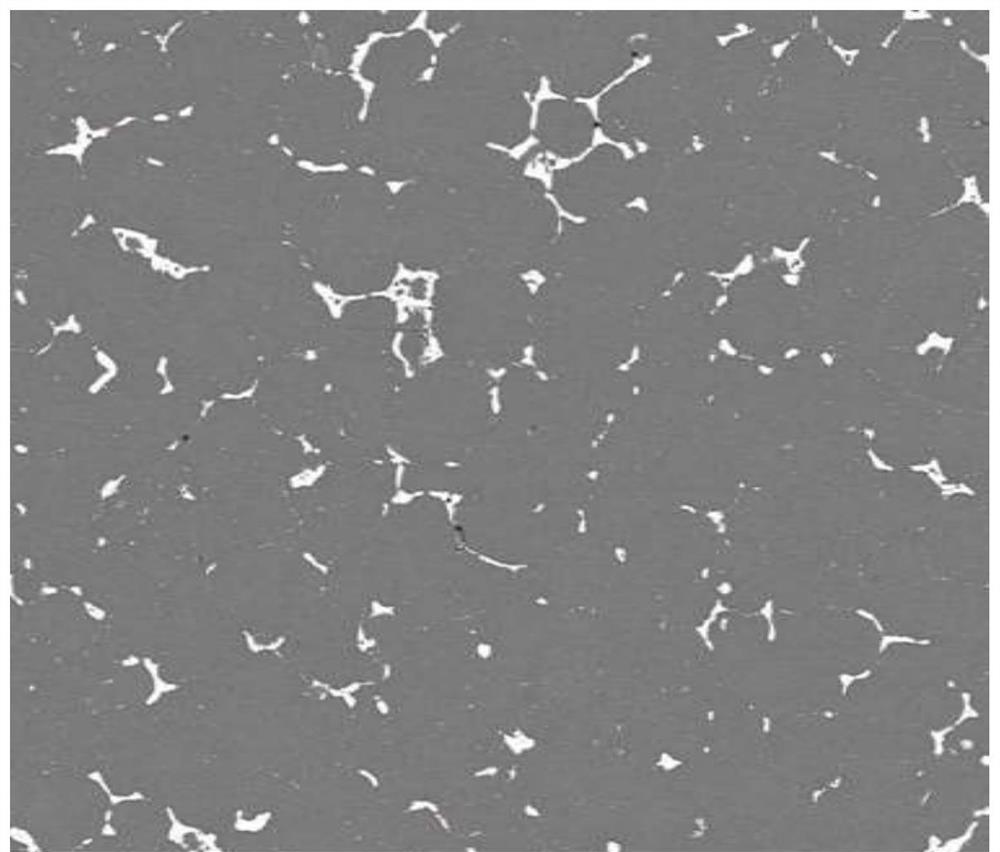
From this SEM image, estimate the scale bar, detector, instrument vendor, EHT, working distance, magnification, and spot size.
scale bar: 200000 nm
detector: DualBSD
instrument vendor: FEI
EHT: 20 kV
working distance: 10.8 mm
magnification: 0.5 K X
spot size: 6